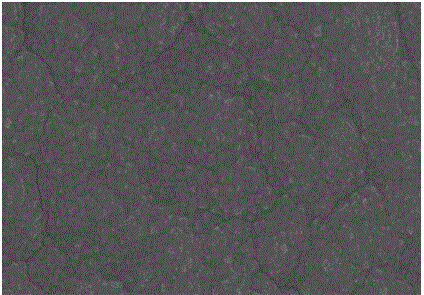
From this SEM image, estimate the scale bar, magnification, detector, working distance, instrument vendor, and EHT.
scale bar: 500 nm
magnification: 100 K X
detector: SE(U)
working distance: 16.7 mm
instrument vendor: Hitachi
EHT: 15 kV